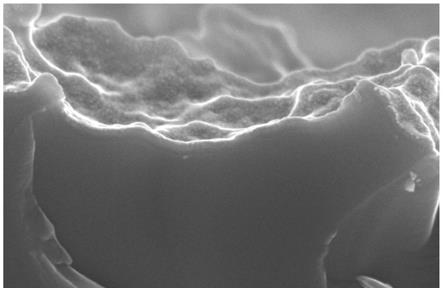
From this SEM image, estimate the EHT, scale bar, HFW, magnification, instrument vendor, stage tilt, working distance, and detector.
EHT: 5 kV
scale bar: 500 nm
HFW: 1.27 µm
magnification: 100 K X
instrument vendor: Thermo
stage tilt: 0°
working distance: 3.7 mm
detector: TLD